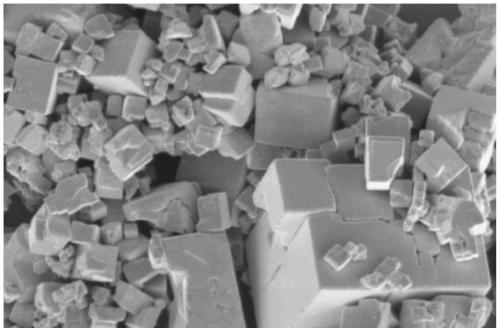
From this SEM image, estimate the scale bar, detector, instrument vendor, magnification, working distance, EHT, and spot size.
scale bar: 10000 nm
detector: ETD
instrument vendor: FEI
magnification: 20 K X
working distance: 3 mm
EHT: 3 kV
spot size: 4.5